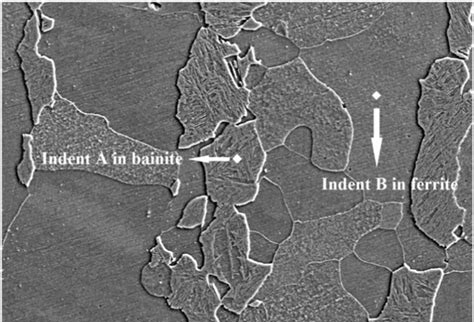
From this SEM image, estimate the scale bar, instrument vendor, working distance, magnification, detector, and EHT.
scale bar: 50000 nm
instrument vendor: Hitachi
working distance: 10.3 mm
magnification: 1 K X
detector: SE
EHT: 20 kV